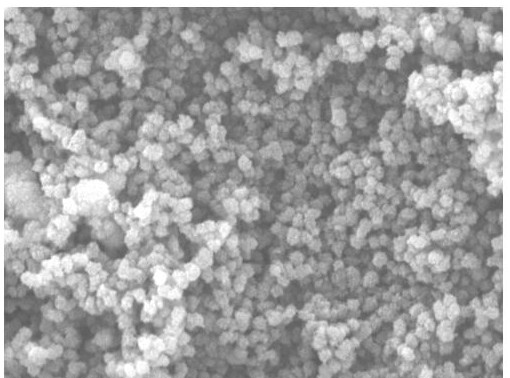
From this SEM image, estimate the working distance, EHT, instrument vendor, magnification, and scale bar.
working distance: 14.11 mm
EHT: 15 kV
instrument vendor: Tescan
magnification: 20 K X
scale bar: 2000 nm